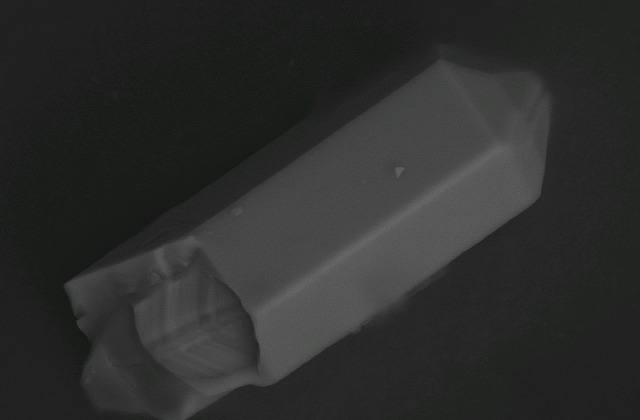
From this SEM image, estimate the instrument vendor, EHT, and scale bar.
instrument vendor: JEOL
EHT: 25 kV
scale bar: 10000 nm